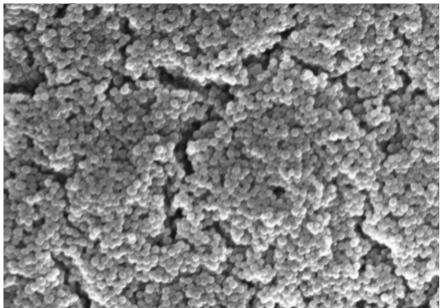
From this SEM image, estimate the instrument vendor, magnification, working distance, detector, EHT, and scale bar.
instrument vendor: Hitachi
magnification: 35 K X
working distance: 12 mm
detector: SE(M)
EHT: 15 kV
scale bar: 1000 nm